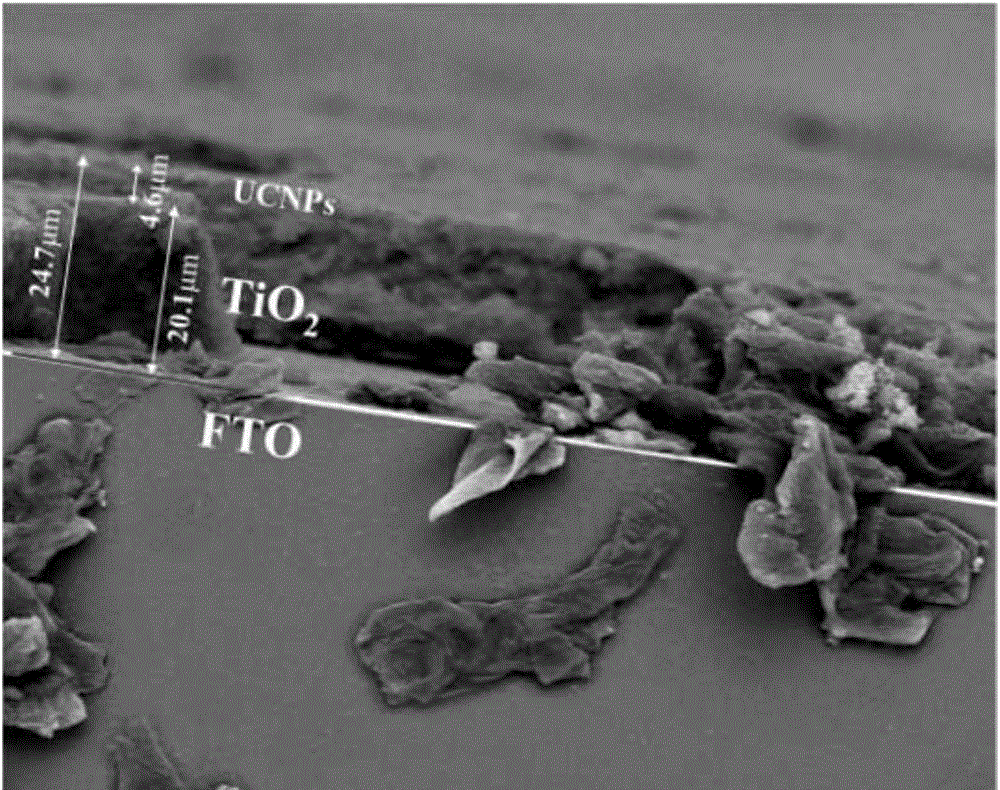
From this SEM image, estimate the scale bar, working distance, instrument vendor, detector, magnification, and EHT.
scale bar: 50000 nm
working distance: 8.7 mm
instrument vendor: FEI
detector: Z Cont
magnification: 2 K X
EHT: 20 kV